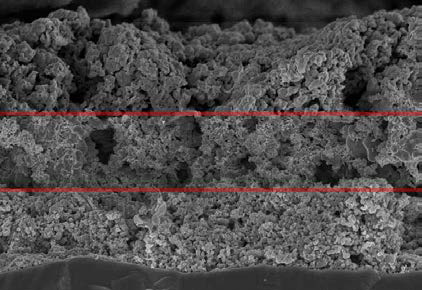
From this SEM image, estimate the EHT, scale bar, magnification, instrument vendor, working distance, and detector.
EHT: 15 kV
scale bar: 10000 nm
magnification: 3 K X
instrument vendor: Hitachi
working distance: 15.7 mm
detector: SE(U)